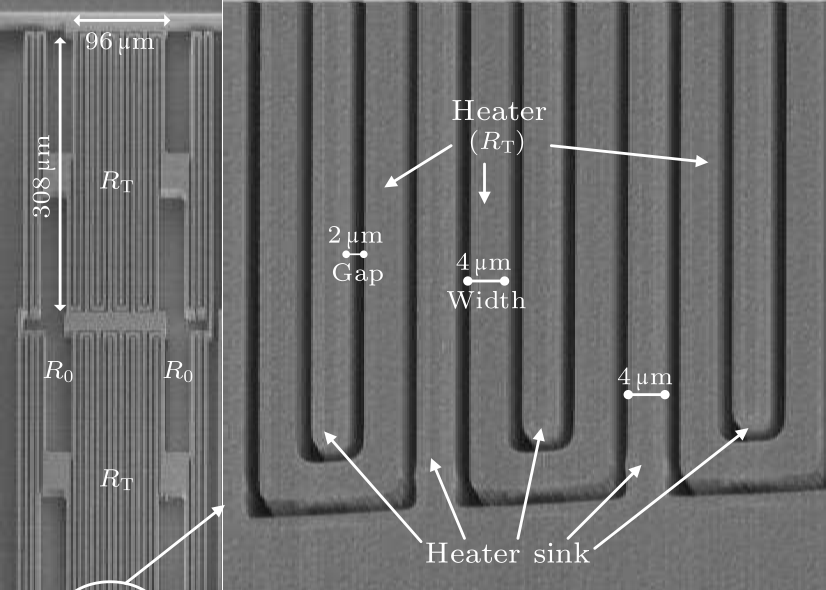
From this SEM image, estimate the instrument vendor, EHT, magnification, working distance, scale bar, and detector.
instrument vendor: Hitachi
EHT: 3 kV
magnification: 1.3 K X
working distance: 8.3 mm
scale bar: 40000 nm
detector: SE(M)